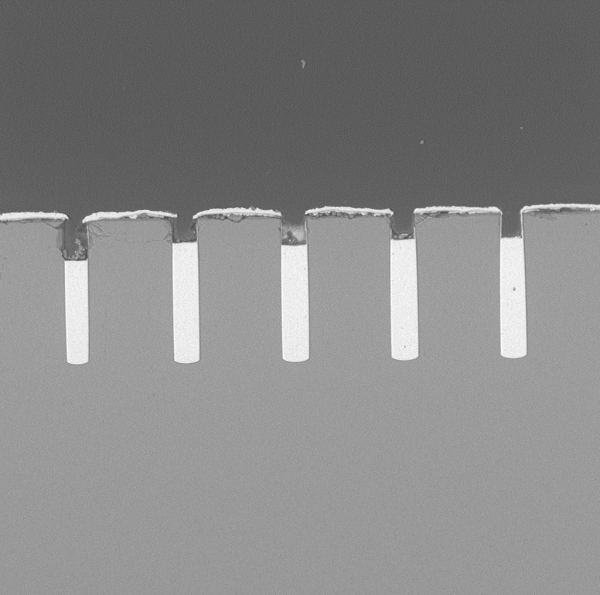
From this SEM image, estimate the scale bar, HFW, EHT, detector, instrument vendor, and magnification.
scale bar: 100000 nm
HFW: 452 µm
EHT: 10 kV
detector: BSD Full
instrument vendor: other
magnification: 0.6 K X